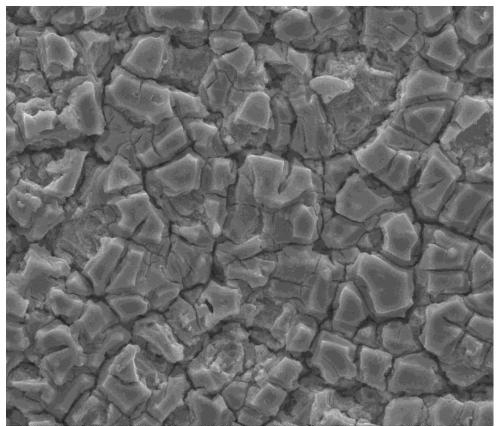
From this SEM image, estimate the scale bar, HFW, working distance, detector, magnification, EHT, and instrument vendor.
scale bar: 10000 nm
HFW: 67.6 µm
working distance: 10.2 mm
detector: ETD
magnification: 2 K X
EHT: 20 kV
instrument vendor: FEI